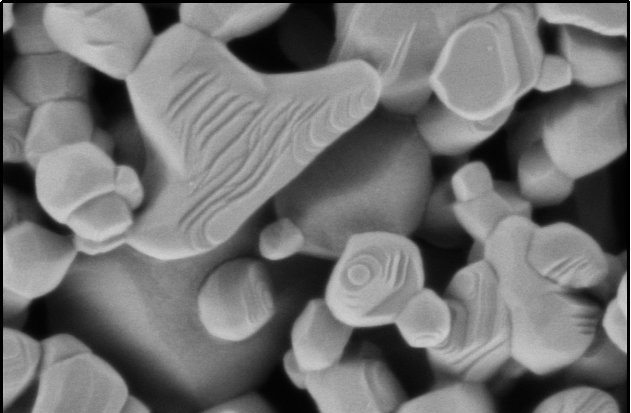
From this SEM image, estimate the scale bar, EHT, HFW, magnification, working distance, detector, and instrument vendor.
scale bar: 500 nm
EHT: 0.5 kV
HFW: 1.27 µm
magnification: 100 K X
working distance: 3.1 mm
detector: T2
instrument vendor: Thermo